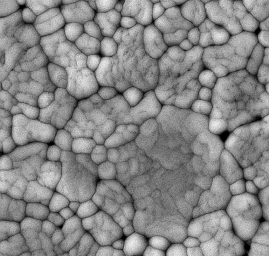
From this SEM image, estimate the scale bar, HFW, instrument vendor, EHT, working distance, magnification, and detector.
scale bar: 1000 nm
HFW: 4.16 µm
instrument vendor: Tescan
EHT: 10 kV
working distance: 9.78 mm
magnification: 50 K X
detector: SE